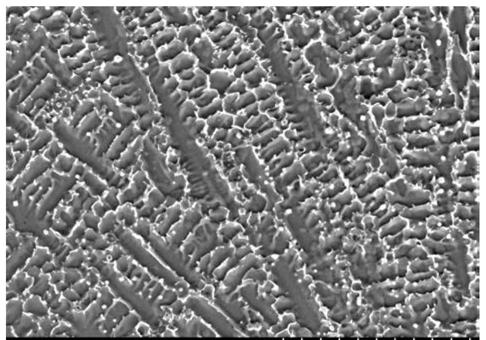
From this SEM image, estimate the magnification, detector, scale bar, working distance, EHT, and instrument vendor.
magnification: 1 K X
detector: SE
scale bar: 50000 nm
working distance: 10.3 mm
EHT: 25 kV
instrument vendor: Hitachi